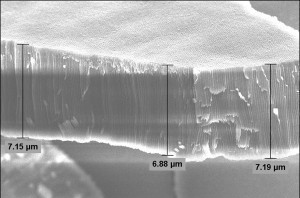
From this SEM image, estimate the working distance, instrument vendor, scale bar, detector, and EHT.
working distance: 9.7 mm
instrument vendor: Zeiss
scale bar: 3000 nm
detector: InLens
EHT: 5 kV